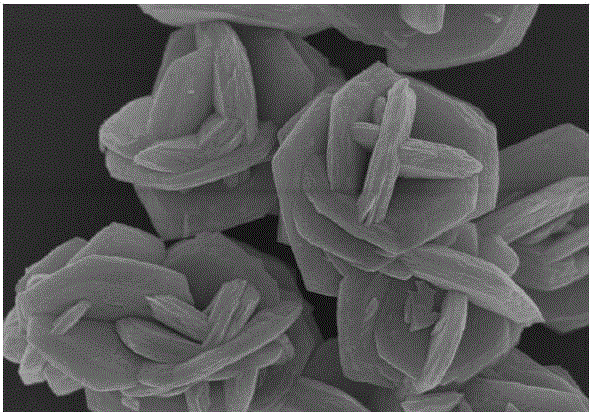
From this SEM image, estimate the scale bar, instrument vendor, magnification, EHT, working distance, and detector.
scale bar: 5000 nm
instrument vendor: Hitachi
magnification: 9 K X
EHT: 15 kV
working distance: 7 mm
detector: SE(M)